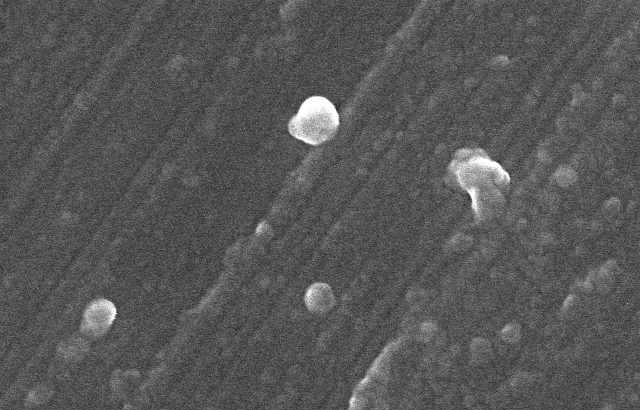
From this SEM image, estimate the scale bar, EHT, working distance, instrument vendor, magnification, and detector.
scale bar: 1000 nm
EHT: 10 kV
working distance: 8.2 mm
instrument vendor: Hitachi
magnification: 50 K X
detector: SE(U)